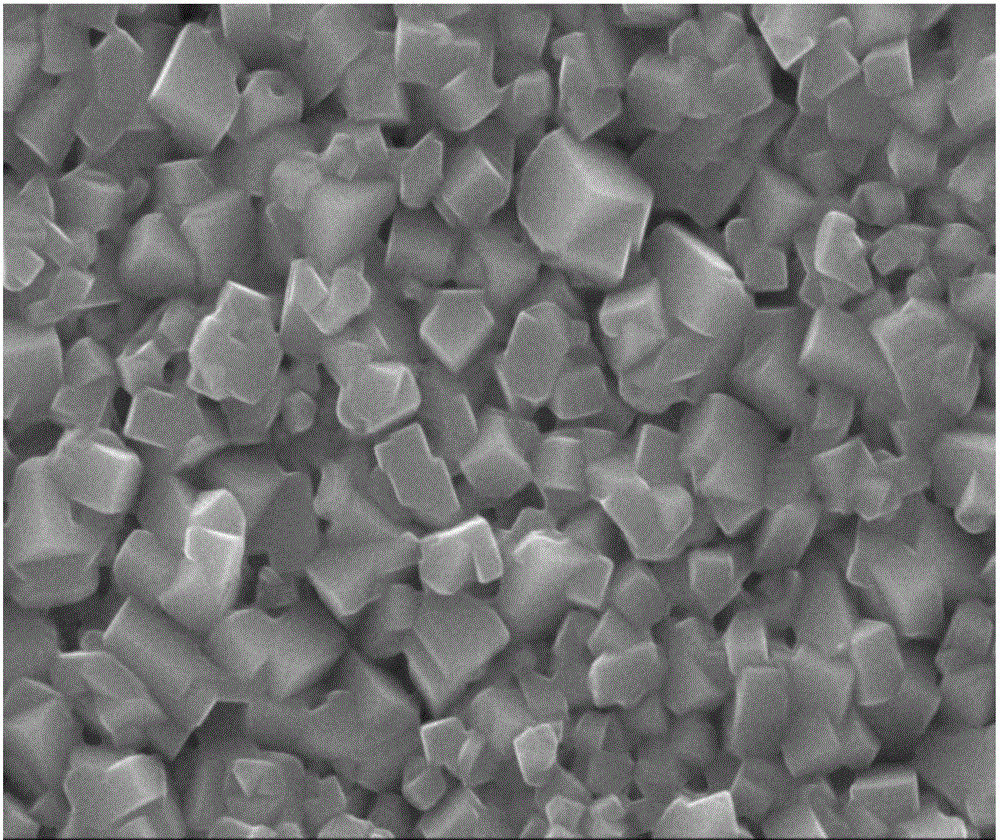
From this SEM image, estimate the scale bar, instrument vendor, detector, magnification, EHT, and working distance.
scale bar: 100 nm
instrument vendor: JEOL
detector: SEI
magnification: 30 K X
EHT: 15 kV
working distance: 6.9 mm